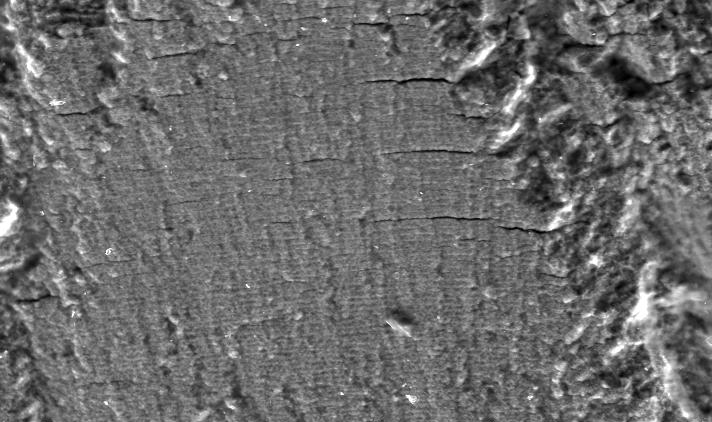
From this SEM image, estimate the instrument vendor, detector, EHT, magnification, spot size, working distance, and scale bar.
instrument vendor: FEI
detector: SE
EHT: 20 kV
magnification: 0.75 K X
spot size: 4.7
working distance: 6.9 mm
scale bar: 20000 nm